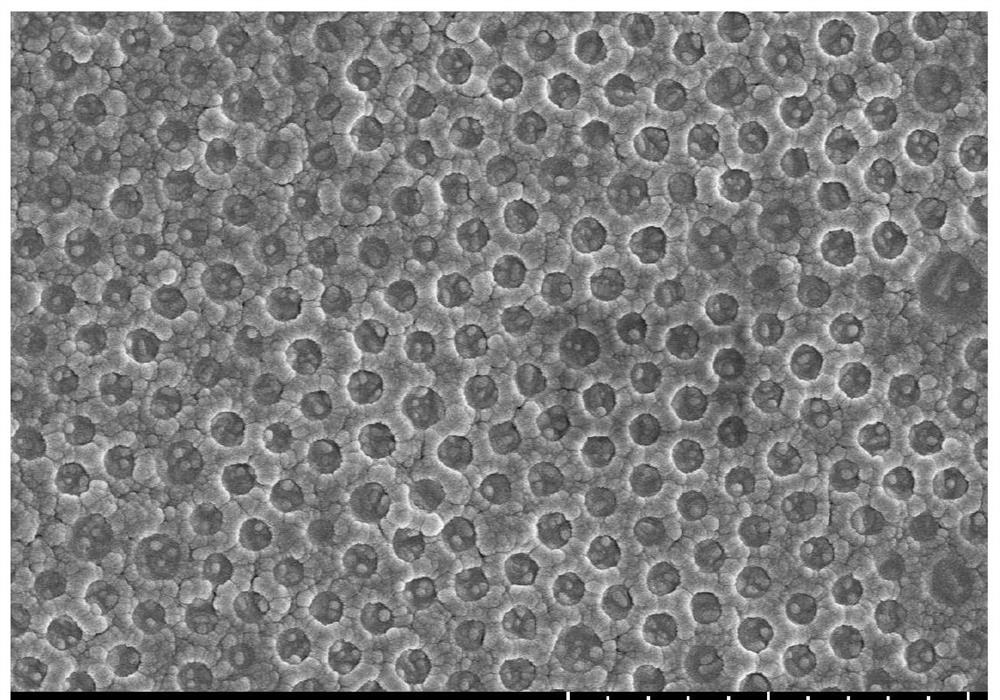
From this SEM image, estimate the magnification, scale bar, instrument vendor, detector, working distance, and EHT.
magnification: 13 K X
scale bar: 4000 nm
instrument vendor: Hitachi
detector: SE(UL)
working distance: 8.3 mm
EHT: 5 kV